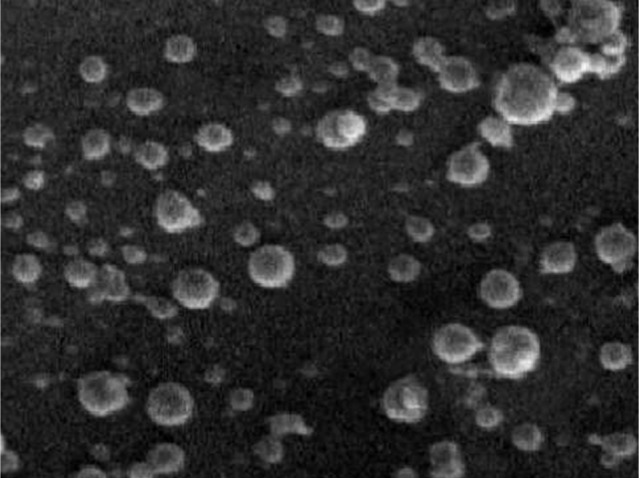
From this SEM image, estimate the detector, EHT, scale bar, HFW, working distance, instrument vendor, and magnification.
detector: SE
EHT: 5 kV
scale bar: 500 nm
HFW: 4.82 µm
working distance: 4.98 mm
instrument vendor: Tescan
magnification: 30 K X